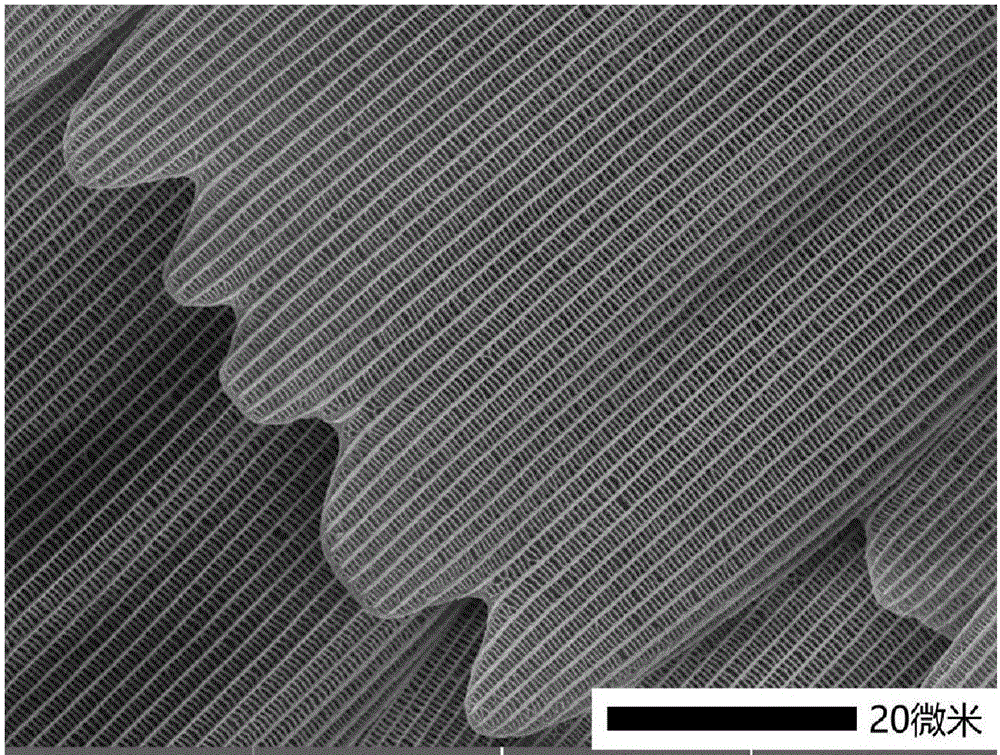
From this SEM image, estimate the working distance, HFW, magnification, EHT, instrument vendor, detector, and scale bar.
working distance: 6.06 mm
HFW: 79.6 µm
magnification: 2.61 K X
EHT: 5 kV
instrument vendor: Tescan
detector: SE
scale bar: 20000 nm